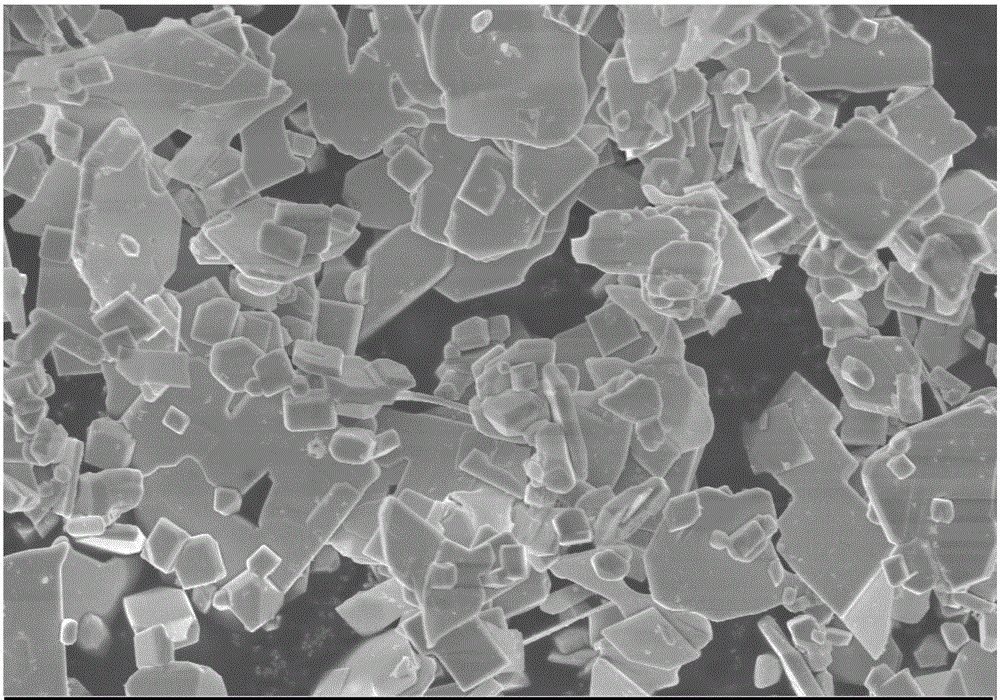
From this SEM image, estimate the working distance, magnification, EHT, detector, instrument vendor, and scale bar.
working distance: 6.7 mm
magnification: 5 K X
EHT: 5 kV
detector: InLens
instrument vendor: Zeiss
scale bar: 2000 nm